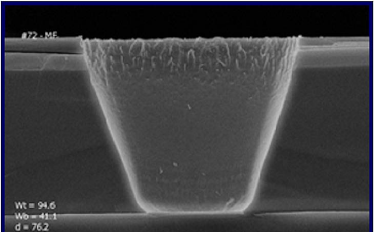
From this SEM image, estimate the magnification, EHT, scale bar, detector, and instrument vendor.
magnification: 1.96 K X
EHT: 25 kV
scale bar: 20000 nm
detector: SE1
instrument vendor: Zeiss